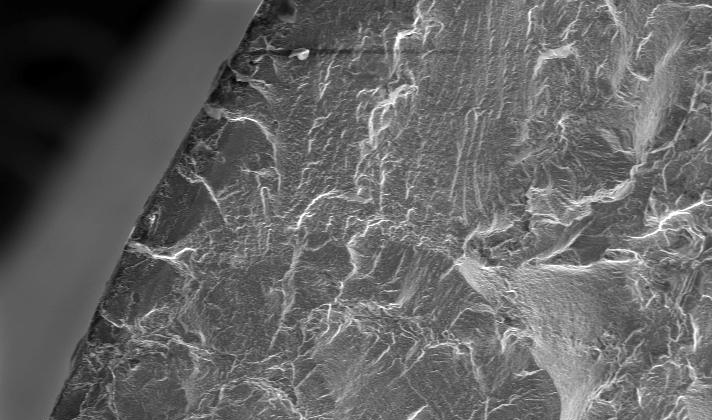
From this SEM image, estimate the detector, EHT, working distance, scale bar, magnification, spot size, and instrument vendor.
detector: SE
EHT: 20 kV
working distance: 20 mm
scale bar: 200000 nm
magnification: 0.125 K X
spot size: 5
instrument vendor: FEI